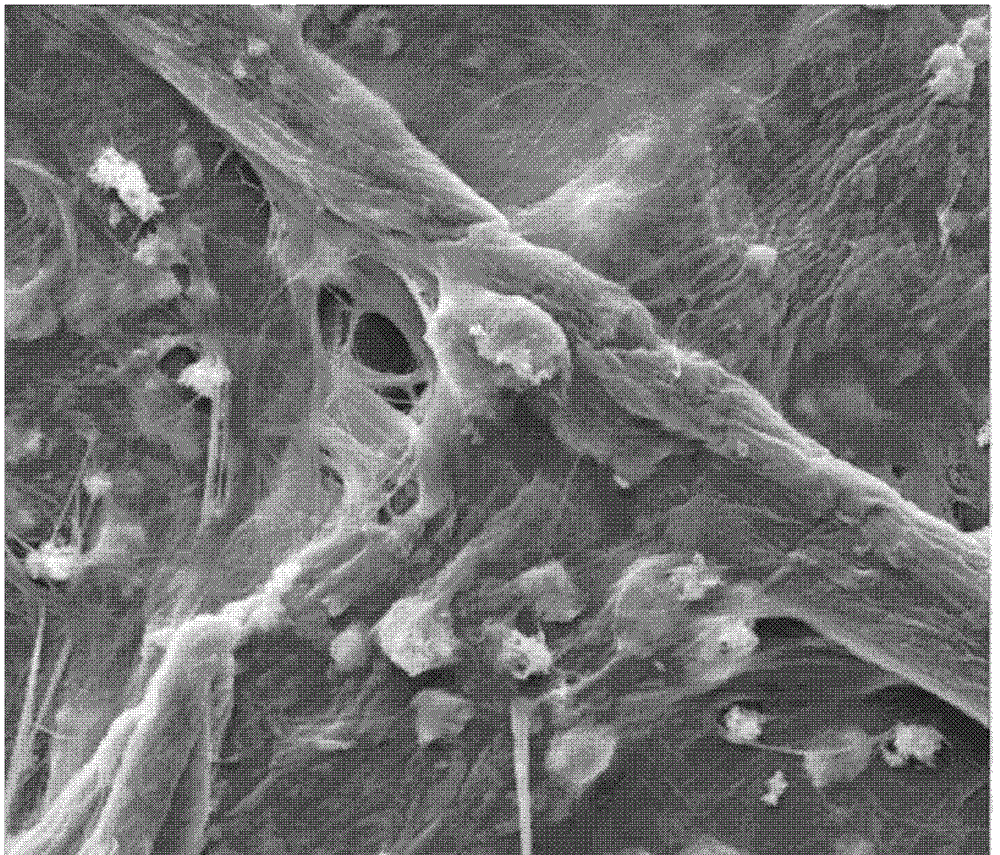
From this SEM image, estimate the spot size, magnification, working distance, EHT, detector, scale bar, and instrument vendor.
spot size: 5.5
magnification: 2 K X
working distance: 9.8 mm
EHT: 12.5 kV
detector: ETD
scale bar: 20000 nm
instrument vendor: FEI